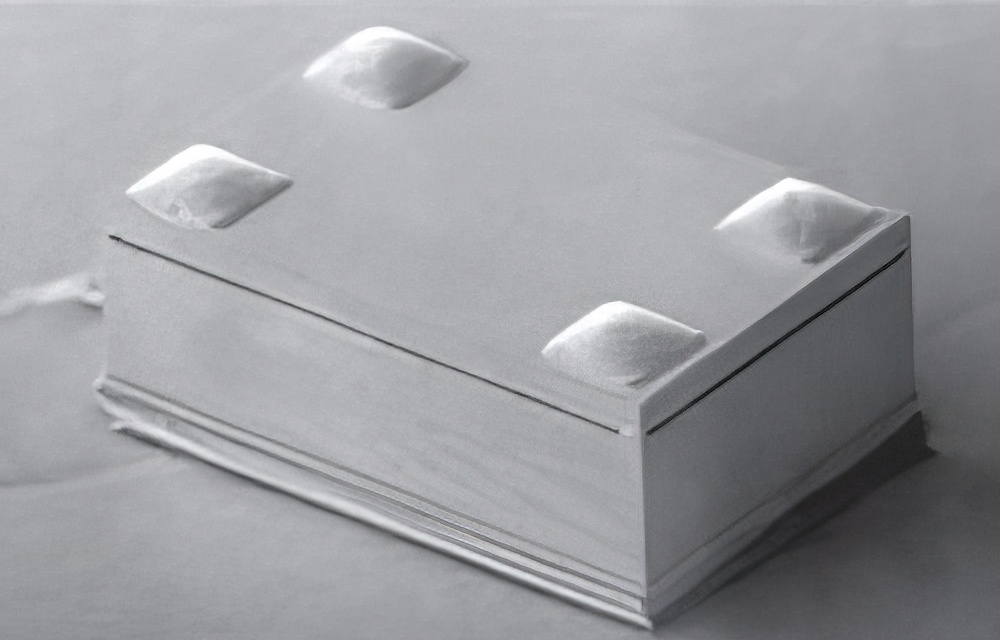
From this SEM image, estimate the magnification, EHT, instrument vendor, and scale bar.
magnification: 0.037 K X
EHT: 10 kV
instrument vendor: JEOL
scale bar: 1000000 nm